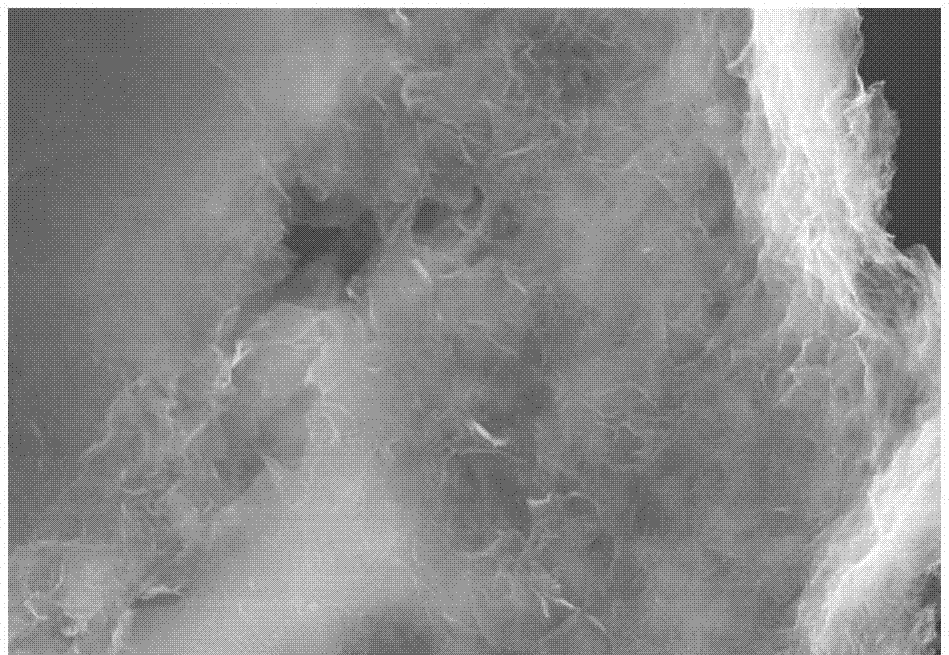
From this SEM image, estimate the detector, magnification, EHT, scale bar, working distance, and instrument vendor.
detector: SE(UL)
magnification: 8 K X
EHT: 15 kV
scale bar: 5000 nm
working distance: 8.8 mm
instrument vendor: Hitachi